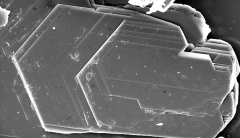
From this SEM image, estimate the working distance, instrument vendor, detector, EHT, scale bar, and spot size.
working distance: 13.2 mm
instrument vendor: FEI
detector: SE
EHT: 20 kV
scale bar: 50000 nm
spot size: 4